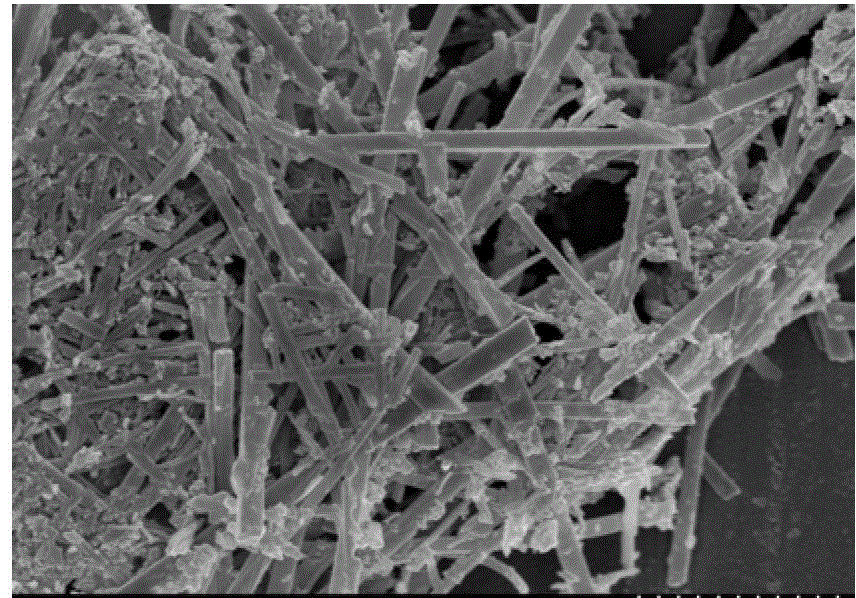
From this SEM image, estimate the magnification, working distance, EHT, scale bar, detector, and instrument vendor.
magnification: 30 K X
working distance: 5.3 mm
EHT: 3 kV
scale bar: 1000 nm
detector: SE(M)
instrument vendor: Hitachi